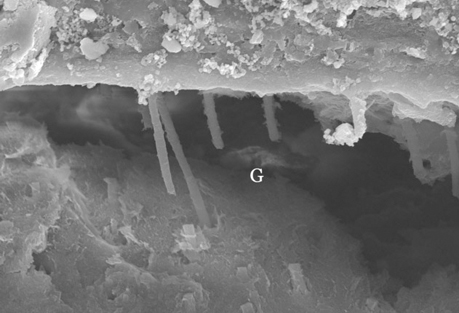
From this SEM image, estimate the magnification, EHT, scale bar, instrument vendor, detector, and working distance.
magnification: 2.5 K X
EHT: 15 kV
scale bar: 20000 nm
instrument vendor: Hitachi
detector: SE(M,HA)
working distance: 14.1 mm